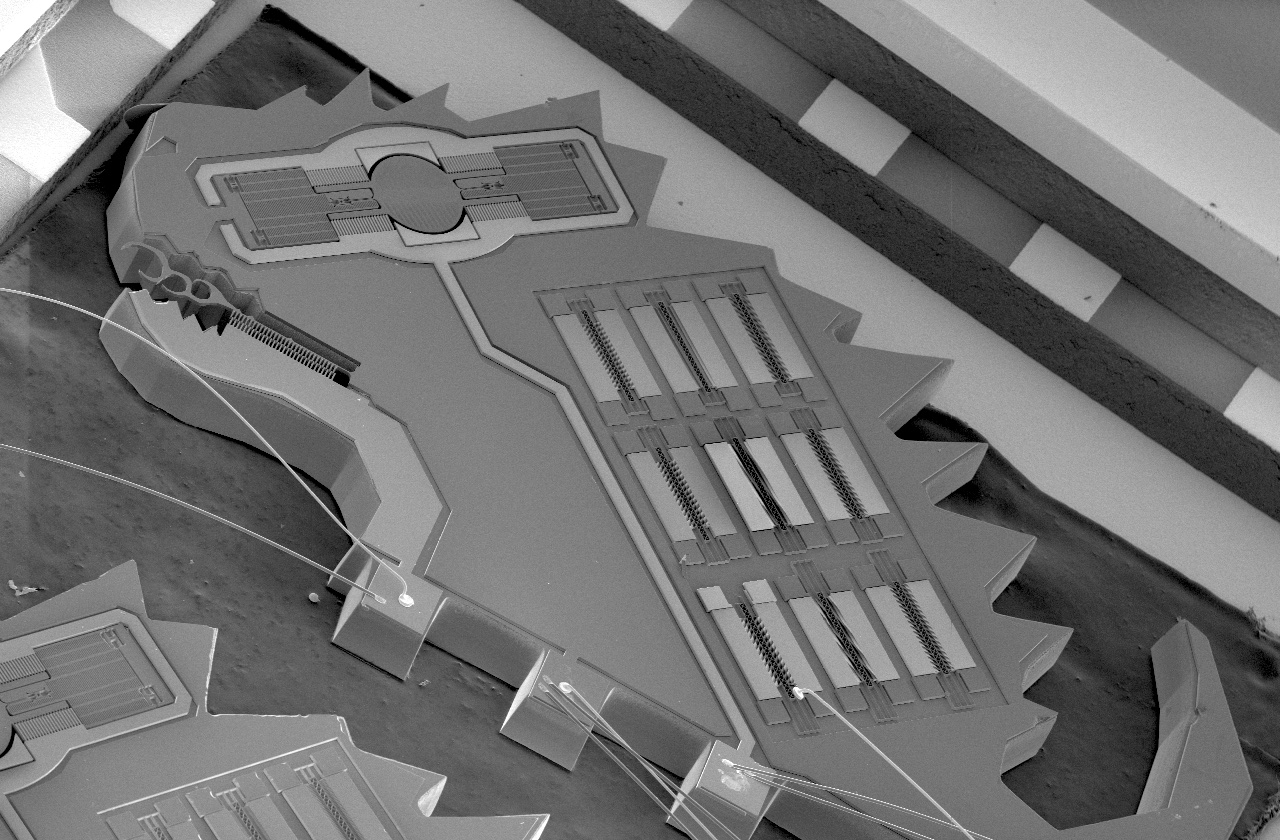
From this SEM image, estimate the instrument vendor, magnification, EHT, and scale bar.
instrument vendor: JEOL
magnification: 0.013 K X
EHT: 15 kV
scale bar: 1000000 nm